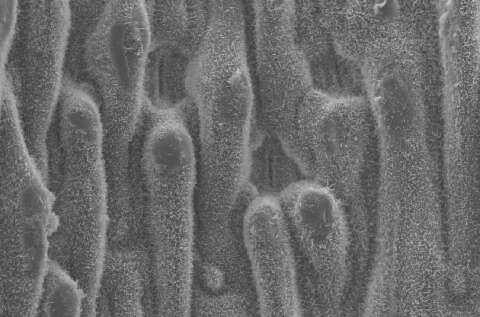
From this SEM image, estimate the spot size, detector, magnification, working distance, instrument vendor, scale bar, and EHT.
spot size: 3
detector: SE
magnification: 0.8 K X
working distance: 10.1 mm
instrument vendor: FEI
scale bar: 20000 nm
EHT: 10 kV